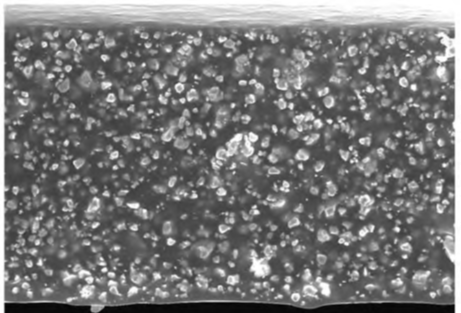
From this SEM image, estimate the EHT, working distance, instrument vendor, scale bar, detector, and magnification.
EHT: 20 kV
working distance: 9.1 mm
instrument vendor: Hitachi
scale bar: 50000 nm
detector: SE(U)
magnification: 0.9 K X